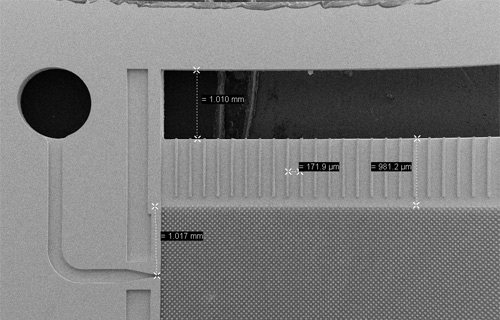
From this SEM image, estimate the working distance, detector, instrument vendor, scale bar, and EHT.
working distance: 19 mm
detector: SE2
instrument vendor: Zeiss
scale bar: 1000000 nm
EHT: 10 kV